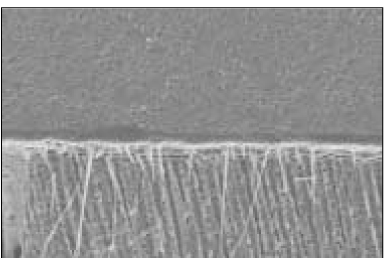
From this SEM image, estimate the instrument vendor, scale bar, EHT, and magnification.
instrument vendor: JEOL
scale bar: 50000 nm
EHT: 15 kV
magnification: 0.3 K X